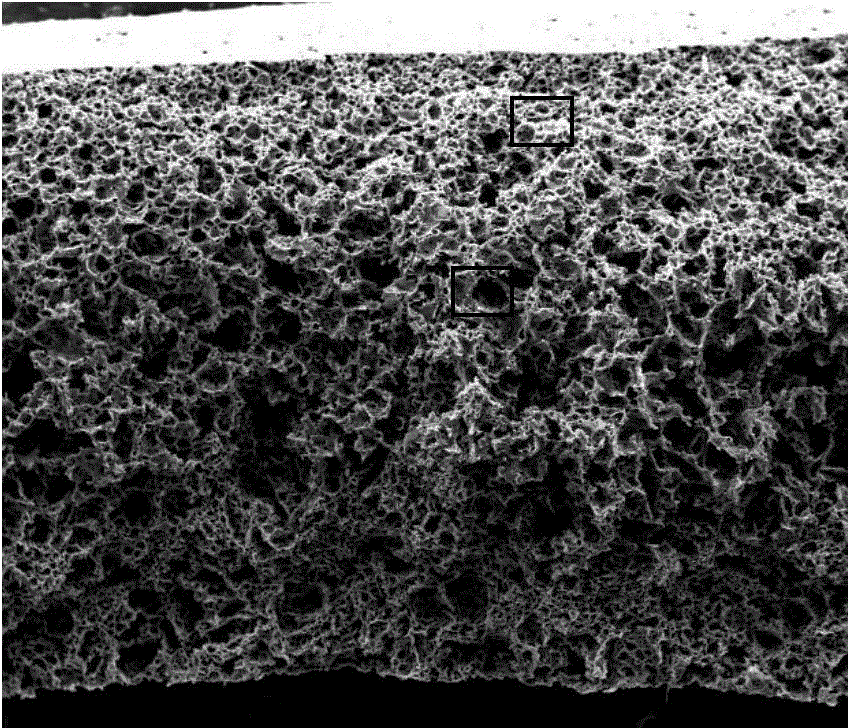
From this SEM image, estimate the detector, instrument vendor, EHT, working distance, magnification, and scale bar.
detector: SE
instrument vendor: FEI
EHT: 20 kV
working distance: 10 mm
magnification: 0.1 K X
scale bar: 1000000 nm